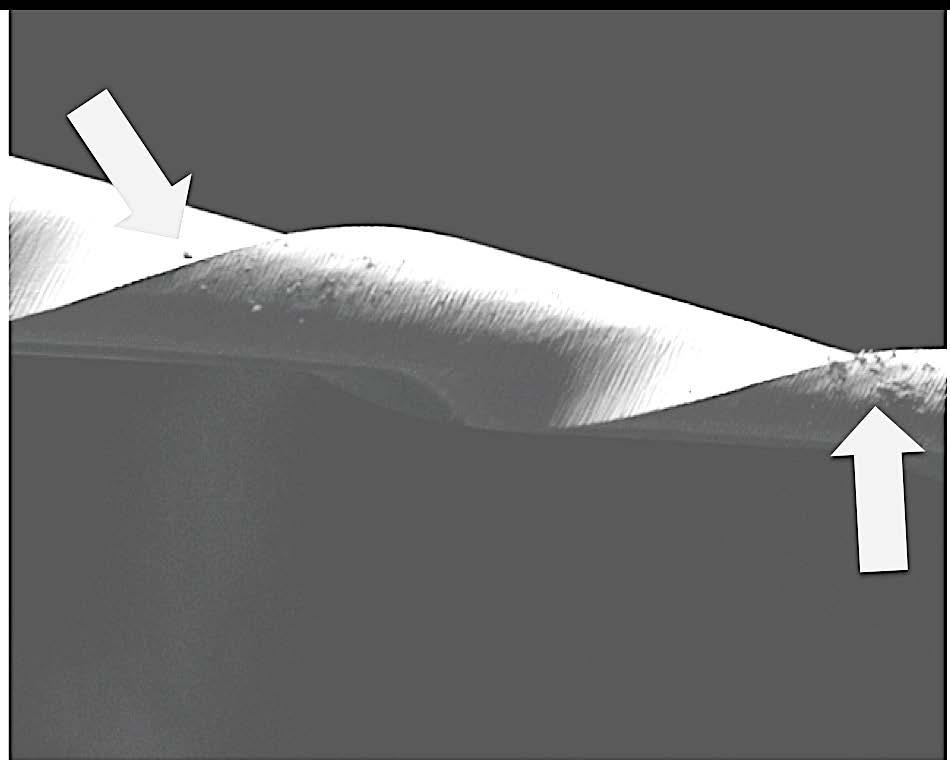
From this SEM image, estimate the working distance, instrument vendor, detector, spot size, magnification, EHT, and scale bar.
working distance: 13.5 mm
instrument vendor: FEI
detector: ETD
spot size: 6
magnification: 0.15 K X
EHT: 20 kV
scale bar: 1000000 nm